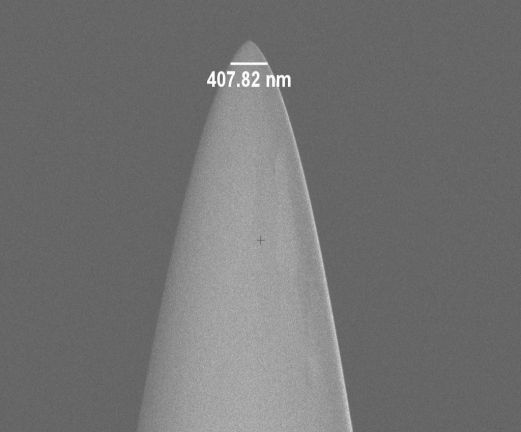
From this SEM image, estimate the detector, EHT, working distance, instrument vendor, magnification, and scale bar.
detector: InLens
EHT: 2 kV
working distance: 4.8 mm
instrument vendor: Zeiss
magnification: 20 K X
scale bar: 2000 nm